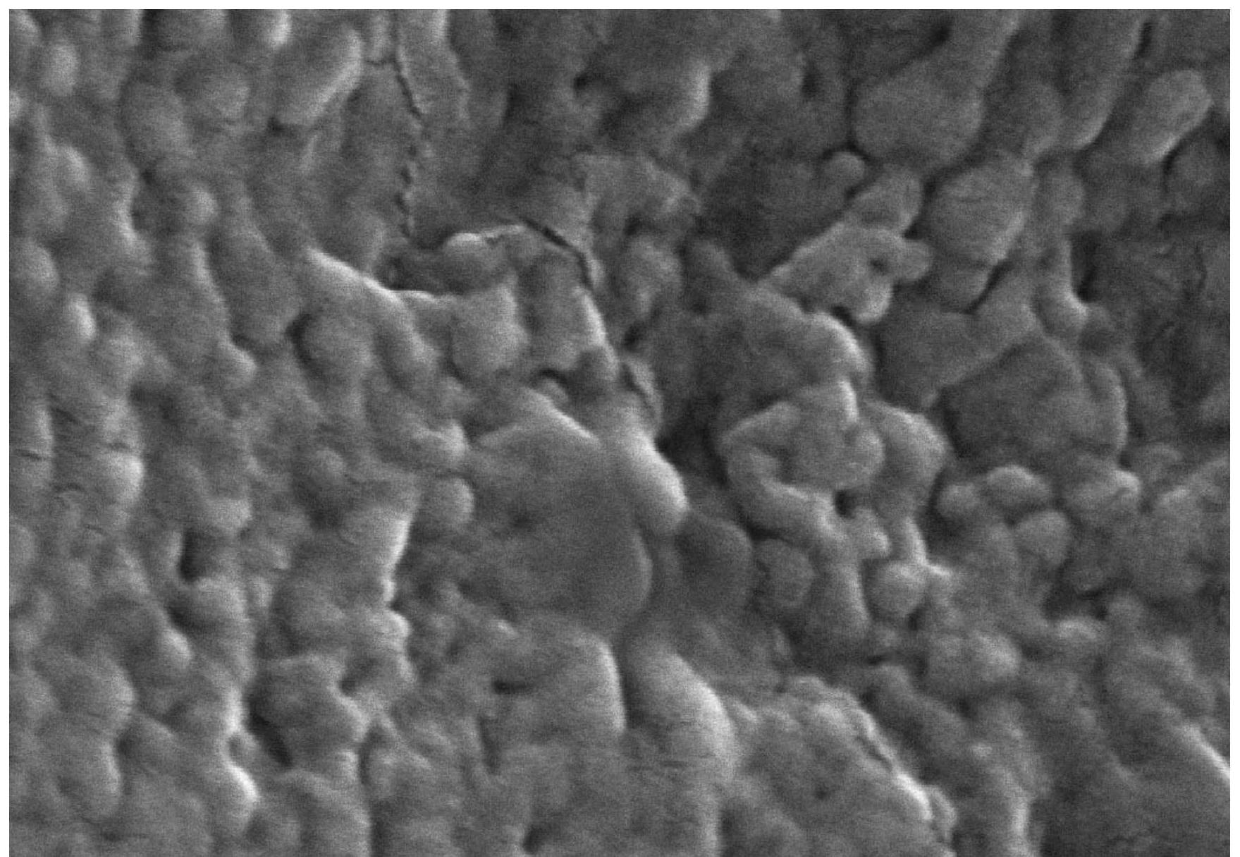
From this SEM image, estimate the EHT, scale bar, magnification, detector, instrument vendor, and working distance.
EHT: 10 kV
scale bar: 300 nm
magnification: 150 K X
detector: SE(M)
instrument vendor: Hitachi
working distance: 8.5 mm